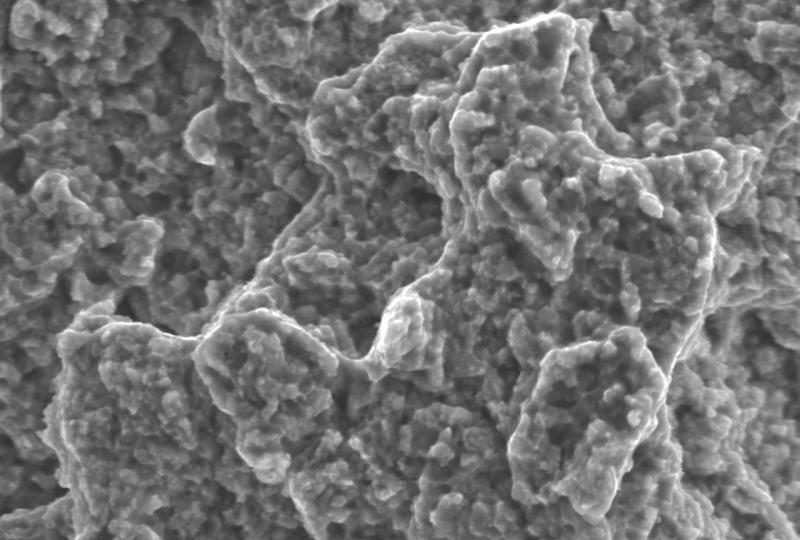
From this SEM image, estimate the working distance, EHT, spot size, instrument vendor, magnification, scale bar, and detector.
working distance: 10 mm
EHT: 20 kV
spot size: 55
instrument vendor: JEOL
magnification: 3 K X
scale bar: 5000 nm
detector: SEI